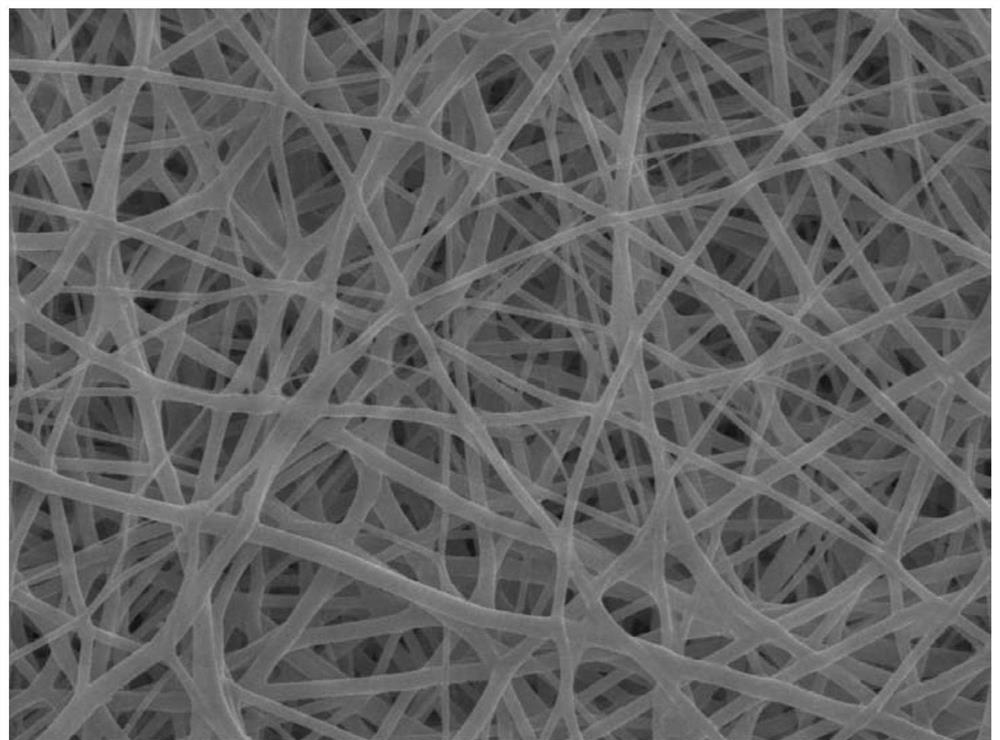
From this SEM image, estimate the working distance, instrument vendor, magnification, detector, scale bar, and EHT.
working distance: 10.1 mm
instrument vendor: JEOL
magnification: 2 K X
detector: SEI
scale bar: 10000 nm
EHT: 15 kV